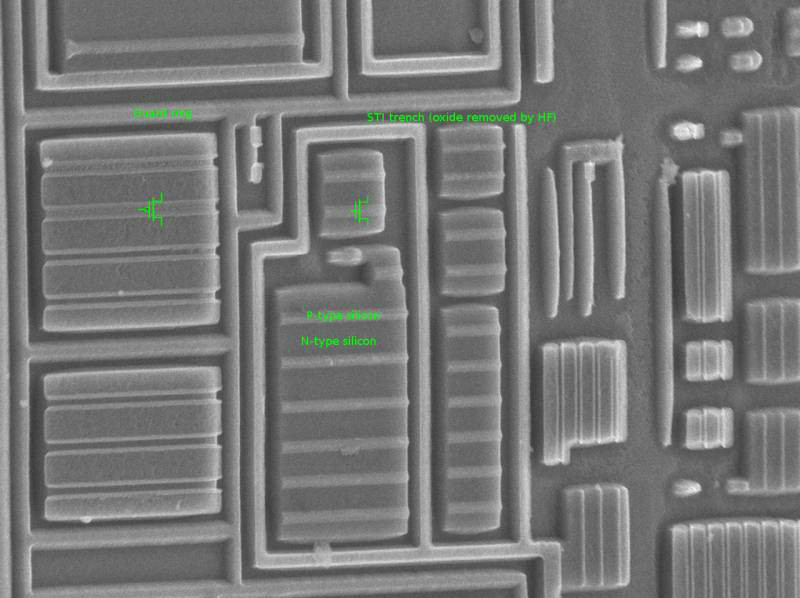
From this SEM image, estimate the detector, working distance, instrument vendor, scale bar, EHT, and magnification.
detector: SEI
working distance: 21 mm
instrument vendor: JEOL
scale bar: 1000 nm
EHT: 10 kV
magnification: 4.5 K X